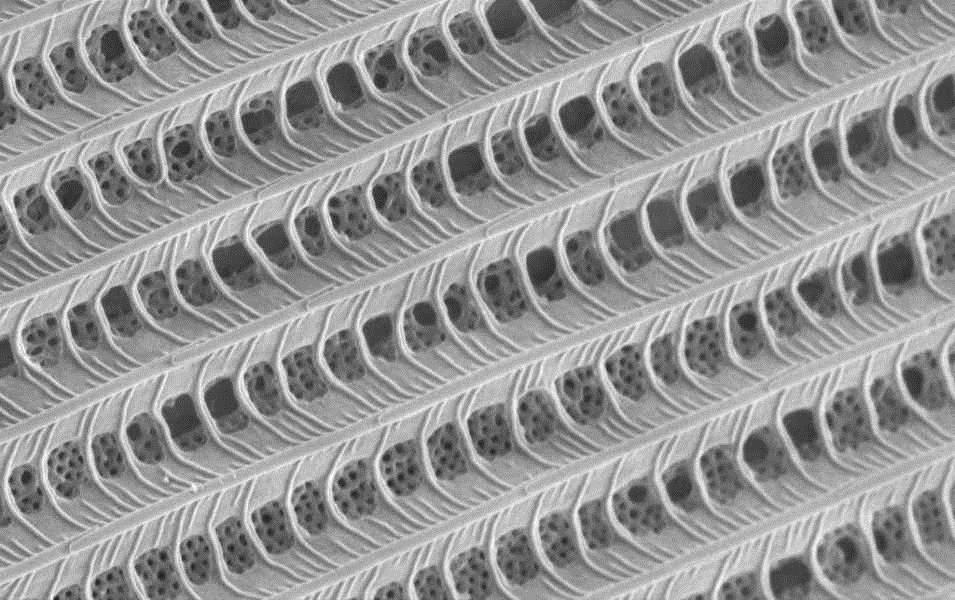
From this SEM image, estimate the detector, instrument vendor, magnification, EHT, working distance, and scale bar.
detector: InLens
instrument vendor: Zeiss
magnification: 20 K X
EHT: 1 kV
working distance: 3 mm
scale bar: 1000 nm